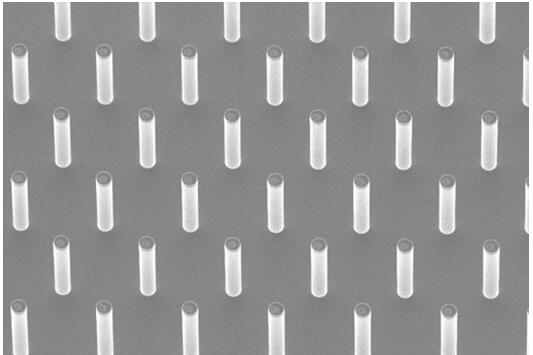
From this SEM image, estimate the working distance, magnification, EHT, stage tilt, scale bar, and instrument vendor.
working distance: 8.2 mm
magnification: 0.5 K X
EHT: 5 kV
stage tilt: -10°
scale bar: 10000 nm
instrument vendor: other